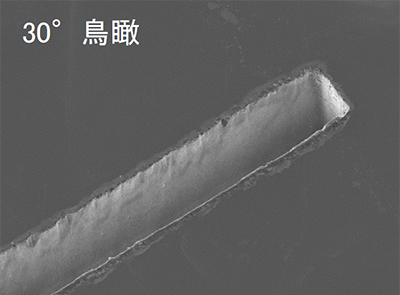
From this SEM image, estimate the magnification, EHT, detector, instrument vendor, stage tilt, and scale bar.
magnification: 0.2 K X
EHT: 10 kV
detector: SEI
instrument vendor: JEOL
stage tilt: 30°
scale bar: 100000 nm